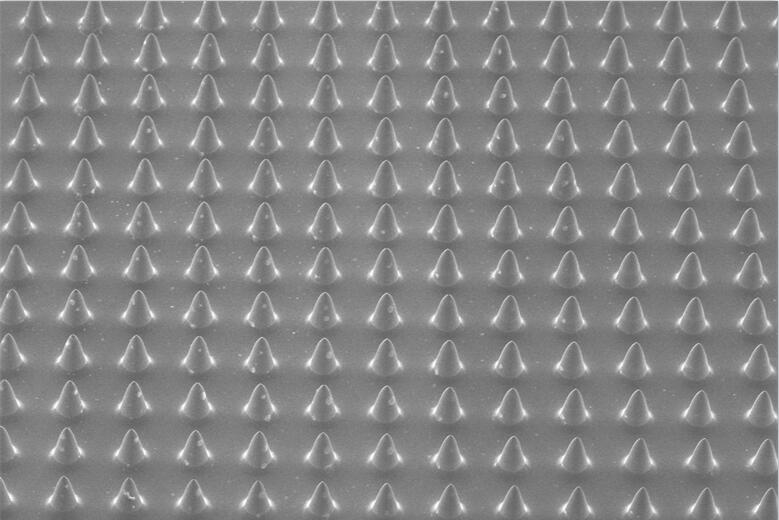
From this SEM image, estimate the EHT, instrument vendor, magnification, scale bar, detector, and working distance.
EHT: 10 kV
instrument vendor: Zeiss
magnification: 0.016 K X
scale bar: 1000000 nm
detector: SE1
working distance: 27.5 mm